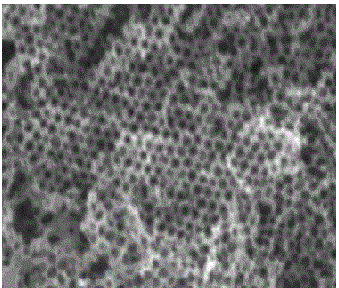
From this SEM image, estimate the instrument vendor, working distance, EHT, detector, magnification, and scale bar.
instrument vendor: Hitachi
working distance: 10.7 mm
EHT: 15 kV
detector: SE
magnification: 20 K X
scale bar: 2000 nm